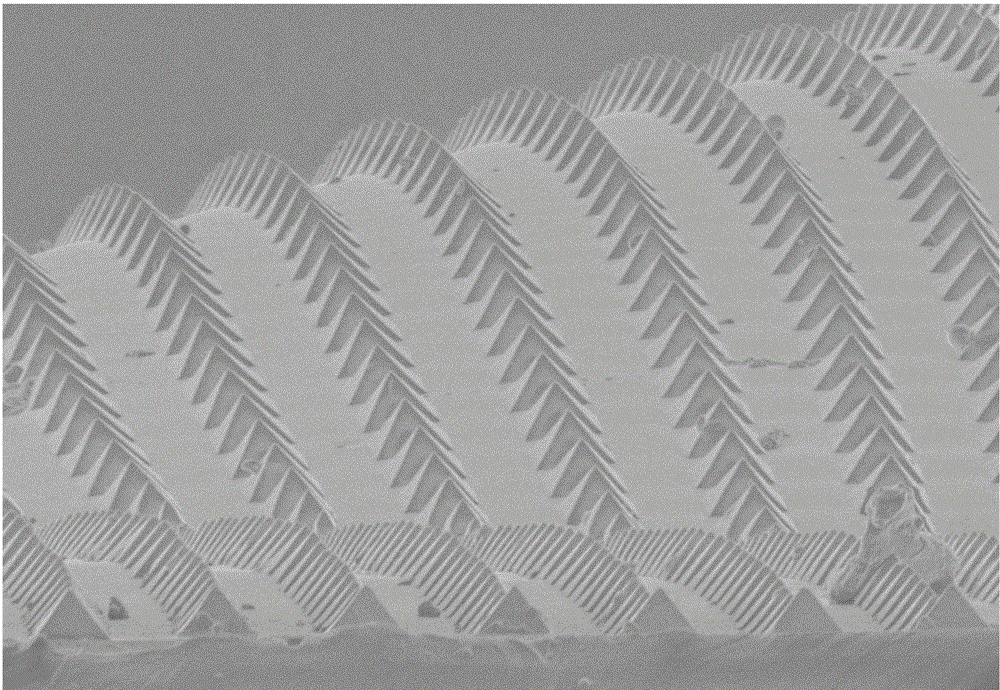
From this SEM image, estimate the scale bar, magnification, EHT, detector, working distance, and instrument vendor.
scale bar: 50000 nm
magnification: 0.9 K X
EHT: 3 kV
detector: SE(M)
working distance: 6.6 mm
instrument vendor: Hitachi